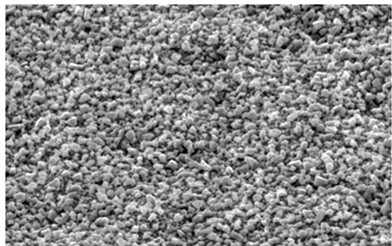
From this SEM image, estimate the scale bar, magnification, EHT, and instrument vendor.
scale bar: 10000 nm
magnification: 2 K X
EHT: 20 kV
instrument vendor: other